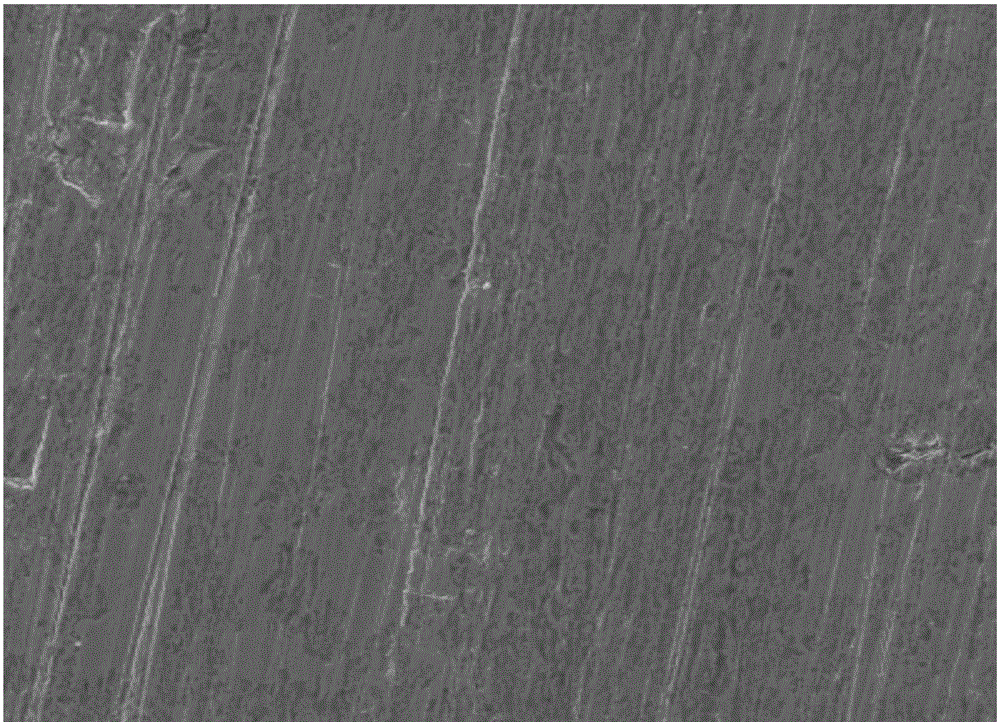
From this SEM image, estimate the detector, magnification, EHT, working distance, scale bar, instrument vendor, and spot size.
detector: ETD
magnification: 0.3 K X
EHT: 20 kV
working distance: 12.6 mm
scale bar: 200000 nm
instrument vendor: FEI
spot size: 4